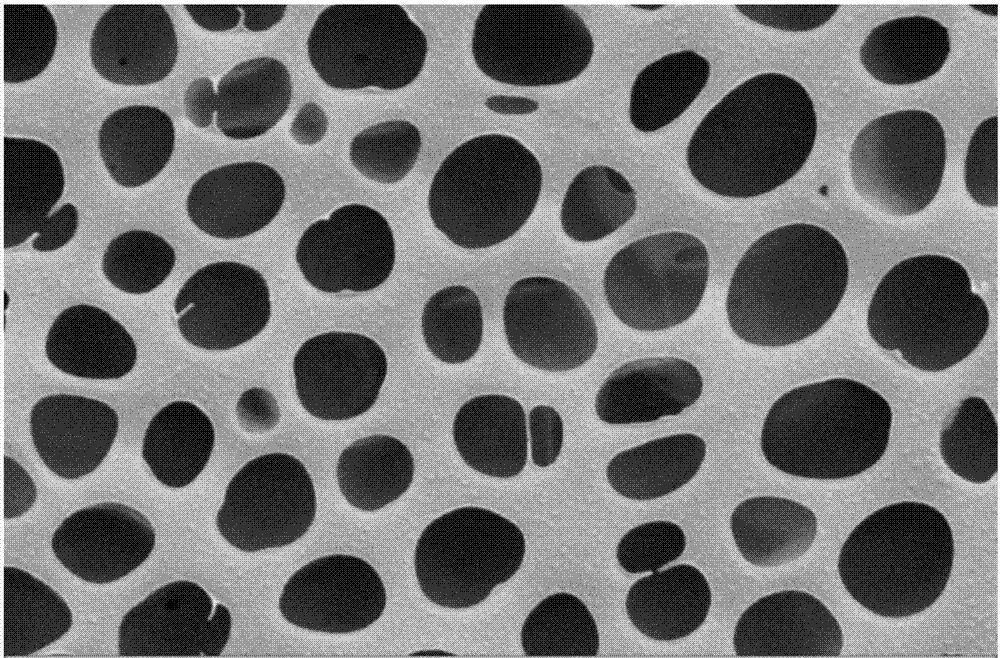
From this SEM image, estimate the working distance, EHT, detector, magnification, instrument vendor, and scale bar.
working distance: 8 mm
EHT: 5 kV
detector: MPSE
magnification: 5 K X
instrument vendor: Zeiss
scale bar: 2000 nm